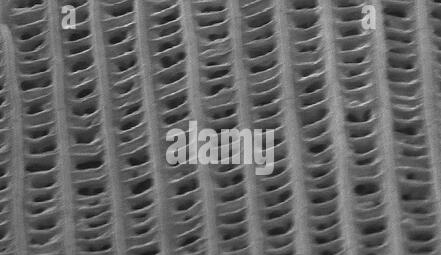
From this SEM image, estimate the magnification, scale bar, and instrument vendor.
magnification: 6.127 K X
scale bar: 5000 nm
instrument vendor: FEI